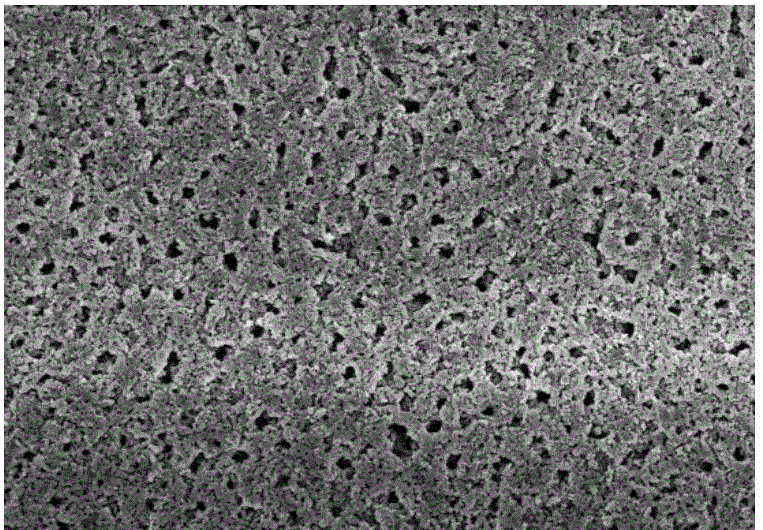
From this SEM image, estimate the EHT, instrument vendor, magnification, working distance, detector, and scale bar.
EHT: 15 kV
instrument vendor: Hitachi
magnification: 1 K X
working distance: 7.2 mm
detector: SE(U)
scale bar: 50000 nm